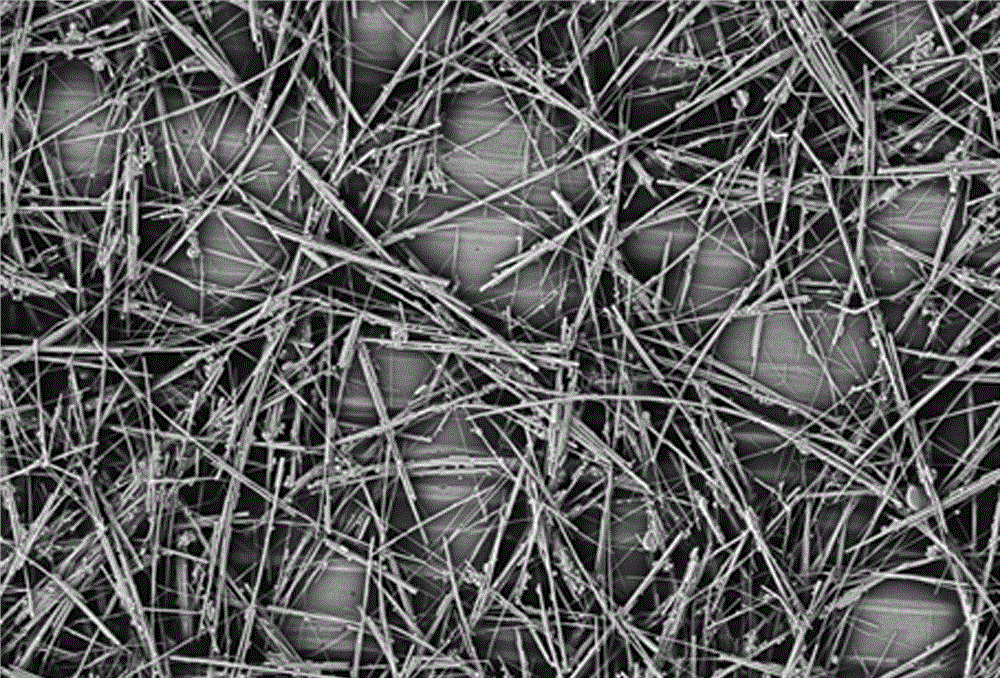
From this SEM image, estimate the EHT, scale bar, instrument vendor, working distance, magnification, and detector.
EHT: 5 kV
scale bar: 2000 nm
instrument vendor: Zeiss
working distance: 7 mm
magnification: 3 K X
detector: SE2